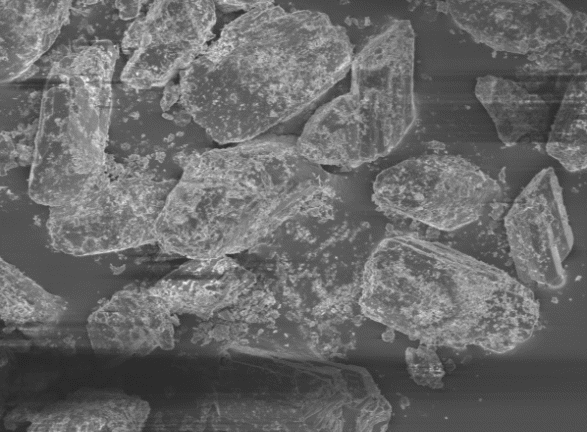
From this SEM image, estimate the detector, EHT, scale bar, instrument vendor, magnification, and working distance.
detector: SEI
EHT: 5 kV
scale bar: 10000 nm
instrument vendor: JEOL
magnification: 0.5 K X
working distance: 16.8 mm